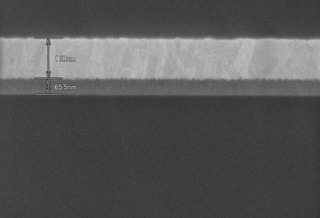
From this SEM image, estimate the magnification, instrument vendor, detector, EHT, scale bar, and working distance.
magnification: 100 K X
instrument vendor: Hitachi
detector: SE(U)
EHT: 3 kV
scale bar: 500 nm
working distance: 3 mm